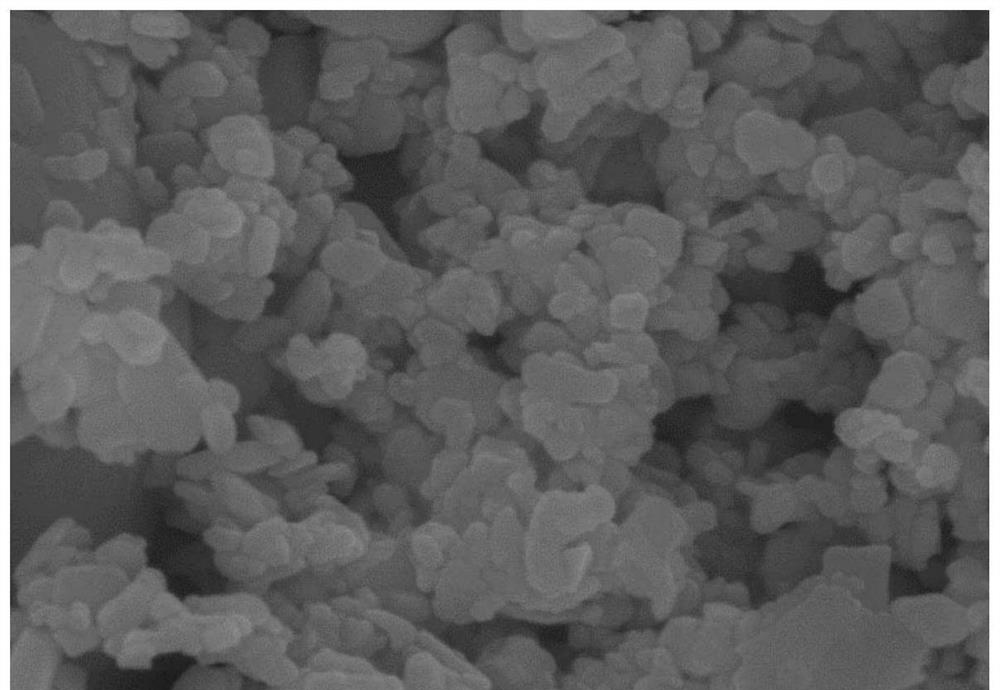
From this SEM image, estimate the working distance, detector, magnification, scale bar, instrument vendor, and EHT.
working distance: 8.2 mm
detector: SE(U)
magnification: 100 K X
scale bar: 500 nm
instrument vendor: Hitachi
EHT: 10 kV